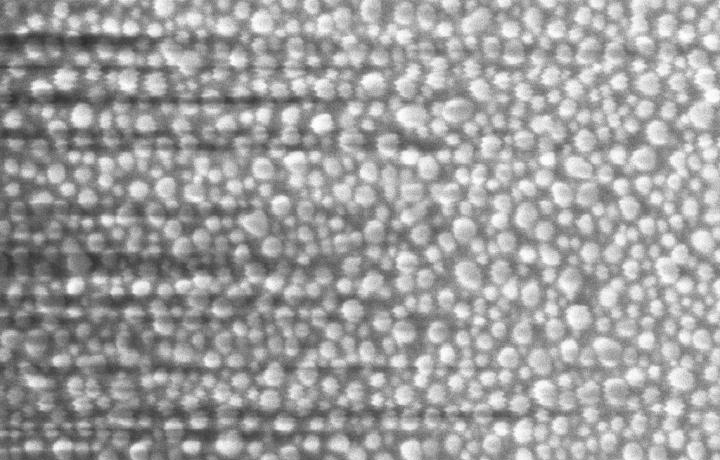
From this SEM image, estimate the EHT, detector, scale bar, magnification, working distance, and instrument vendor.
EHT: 3 kV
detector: InLens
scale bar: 100 nm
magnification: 150 K X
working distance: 3.7 mm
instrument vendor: Zeiss